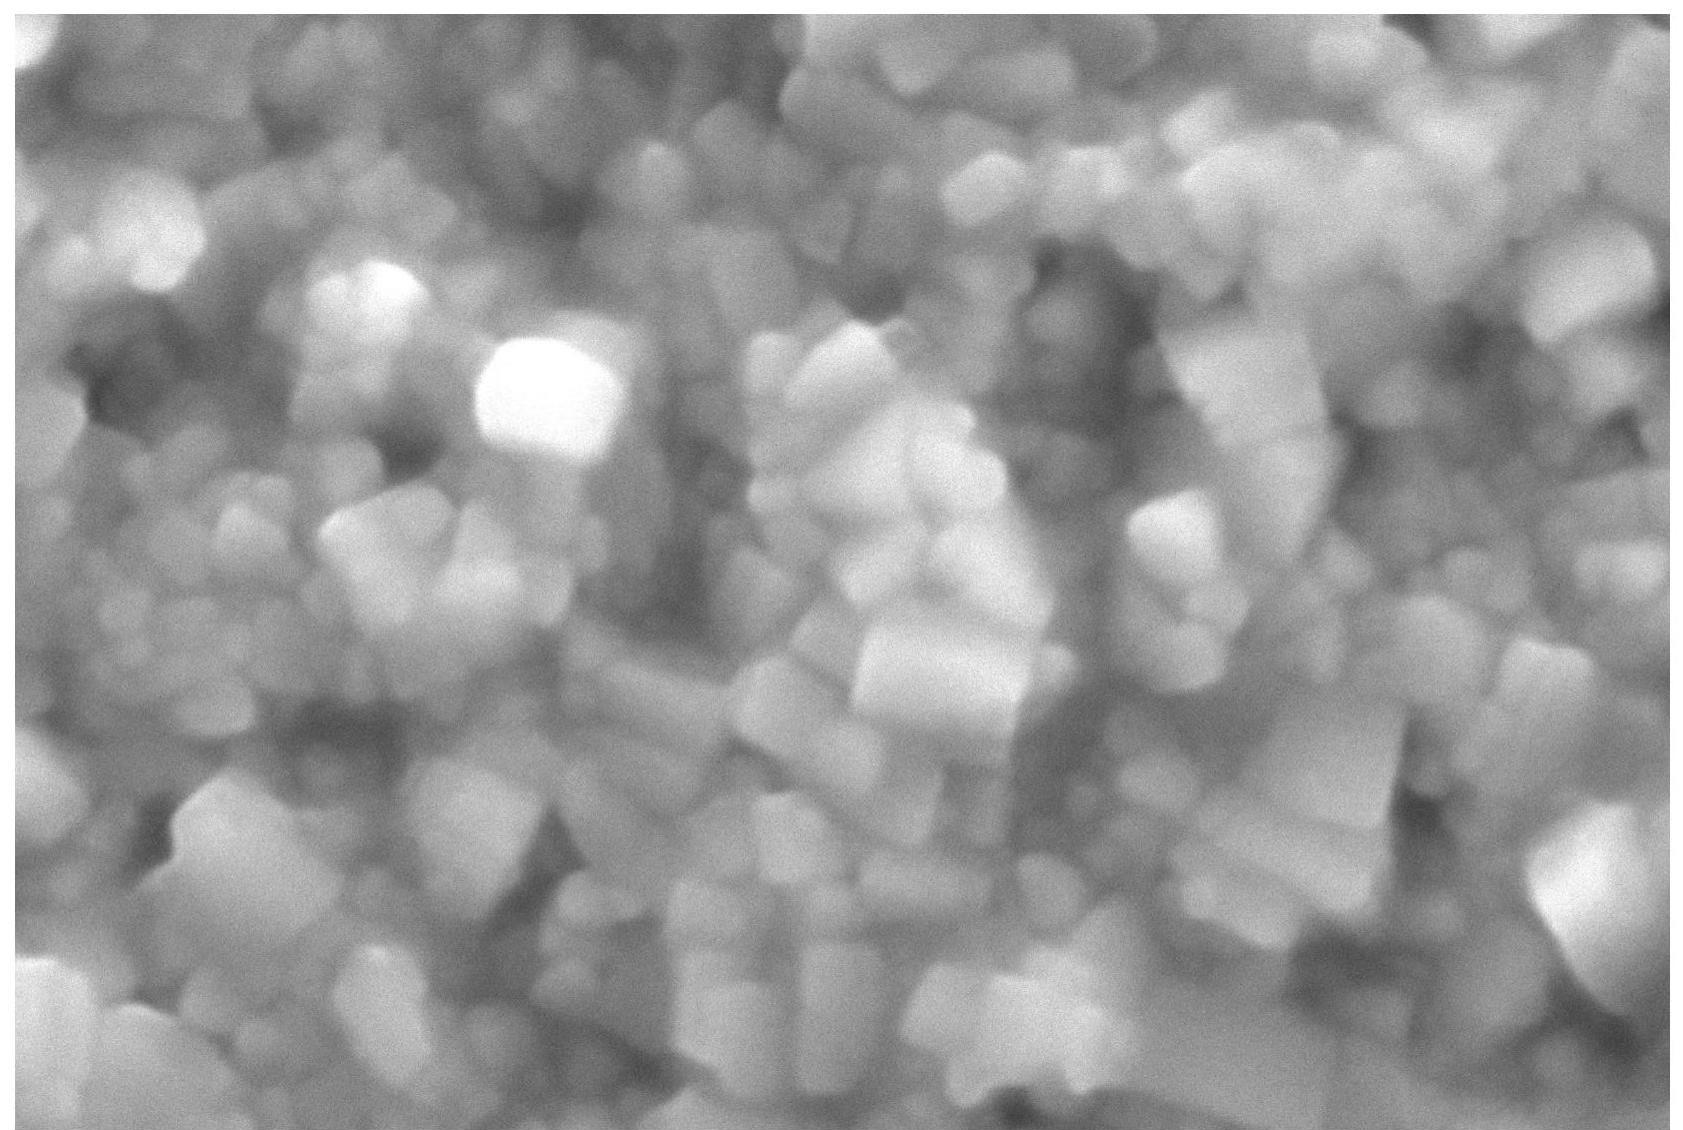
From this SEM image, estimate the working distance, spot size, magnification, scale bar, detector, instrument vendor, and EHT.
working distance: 11 mm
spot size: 30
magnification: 20 K X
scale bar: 1000 nm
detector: SEI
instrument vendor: JEOL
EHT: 15 kV